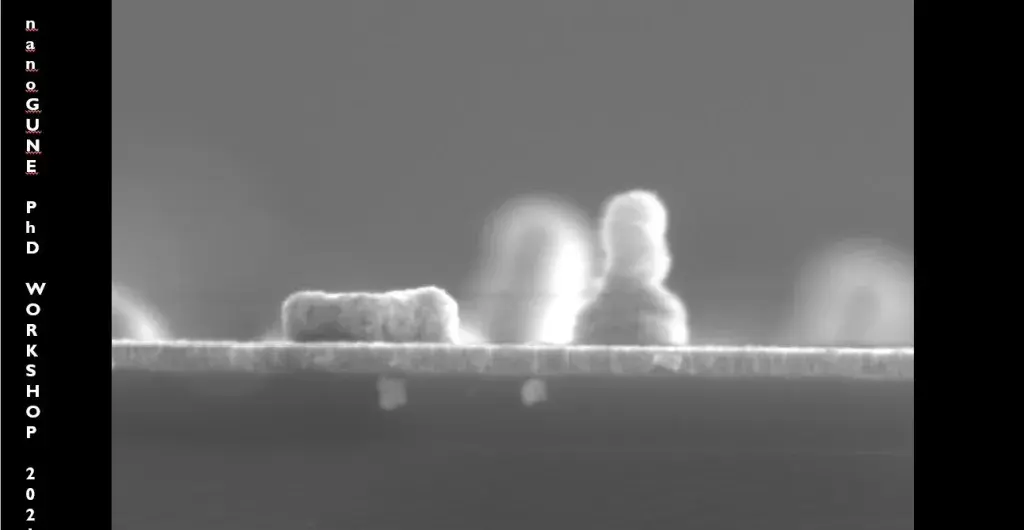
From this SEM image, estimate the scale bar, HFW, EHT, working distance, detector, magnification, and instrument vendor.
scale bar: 500 nm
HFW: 3.19 µm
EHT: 5 kV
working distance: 4 mm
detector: ETD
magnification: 65 K X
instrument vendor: FEI